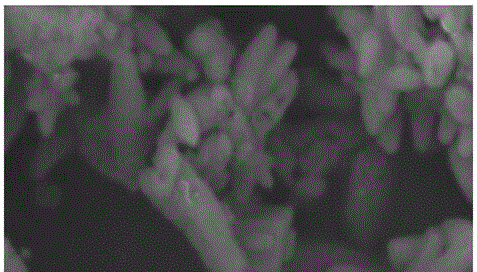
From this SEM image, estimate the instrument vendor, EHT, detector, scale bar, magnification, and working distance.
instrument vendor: Zeiss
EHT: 5 kV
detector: SE2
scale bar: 200 nm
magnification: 25 K X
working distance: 8.5 mm